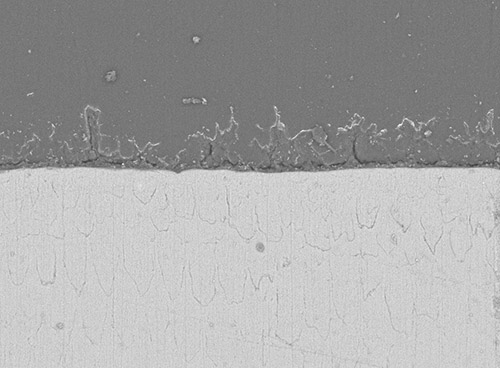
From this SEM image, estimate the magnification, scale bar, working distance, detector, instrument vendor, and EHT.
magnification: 1 K X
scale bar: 10000 nm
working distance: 11.7 mm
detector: COMPO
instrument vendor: JEOL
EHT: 10 kV